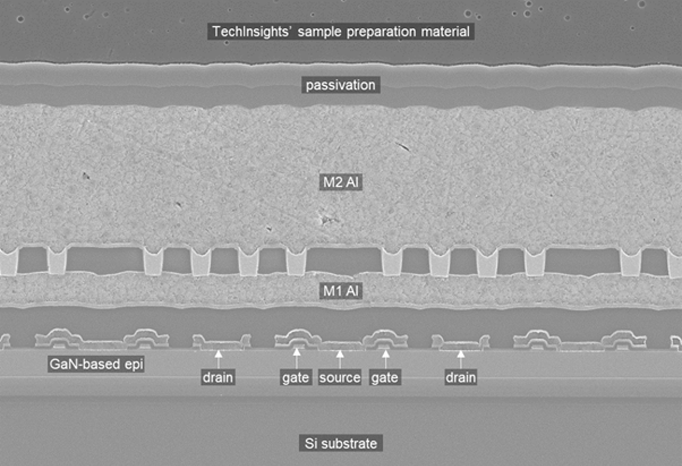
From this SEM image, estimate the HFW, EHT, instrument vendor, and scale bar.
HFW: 19.99 µm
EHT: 5 kV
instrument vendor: Zeiss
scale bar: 2000 nm